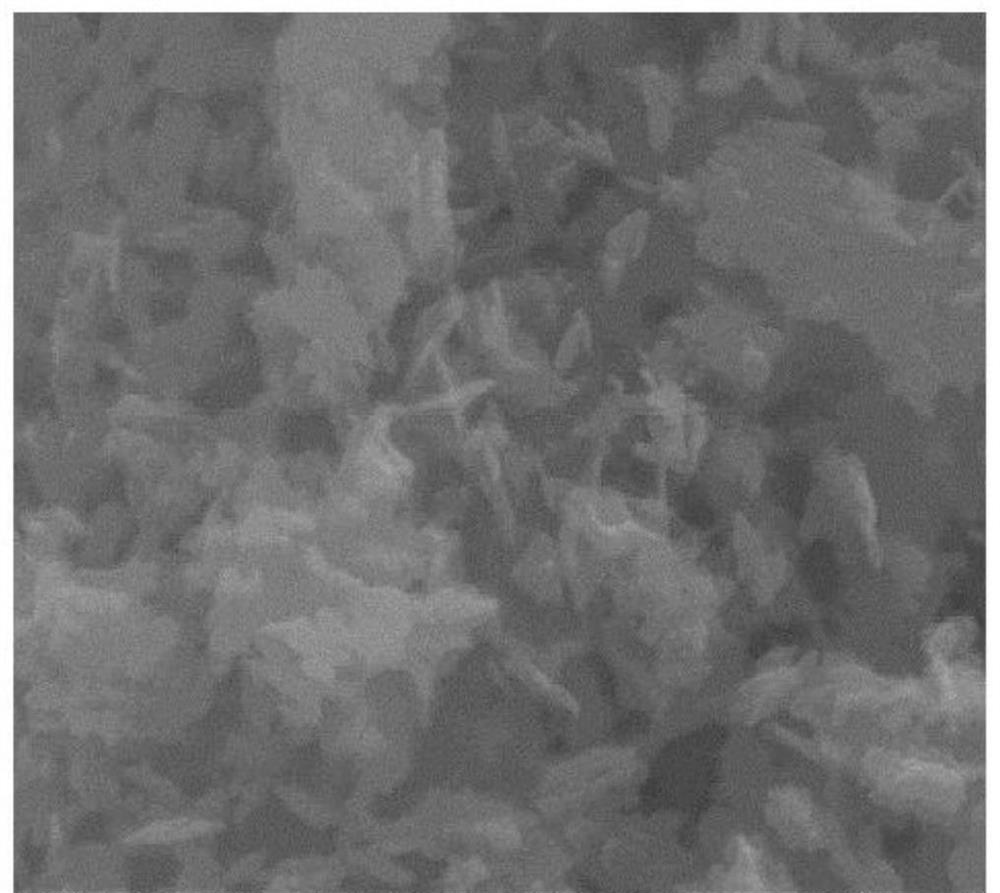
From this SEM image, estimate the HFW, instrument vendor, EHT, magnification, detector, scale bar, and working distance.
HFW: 2.25 µm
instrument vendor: FEI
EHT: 20 kV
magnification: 60 K X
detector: ETD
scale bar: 500 nm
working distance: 9.4 mm